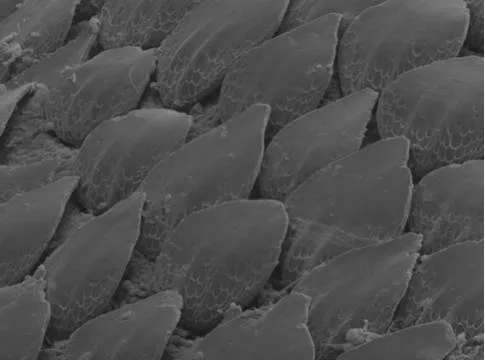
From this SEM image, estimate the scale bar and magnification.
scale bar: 20000 nm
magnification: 0.187 K X